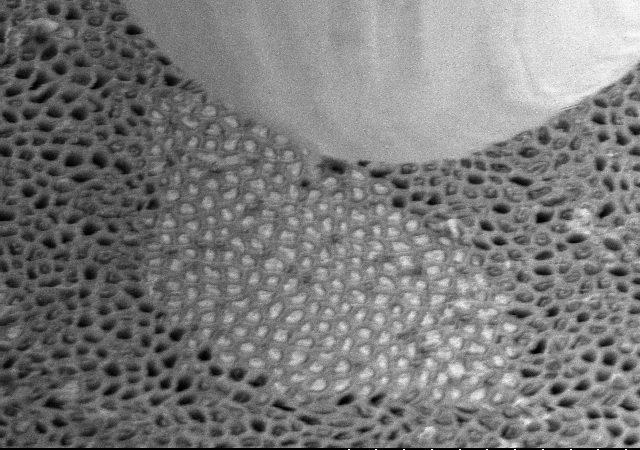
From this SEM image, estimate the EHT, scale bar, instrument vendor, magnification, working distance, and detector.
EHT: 5 kV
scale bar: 5000 nm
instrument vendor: Hitachi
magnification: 11 K X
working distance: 26.5 mm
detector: SE(M,LA80)